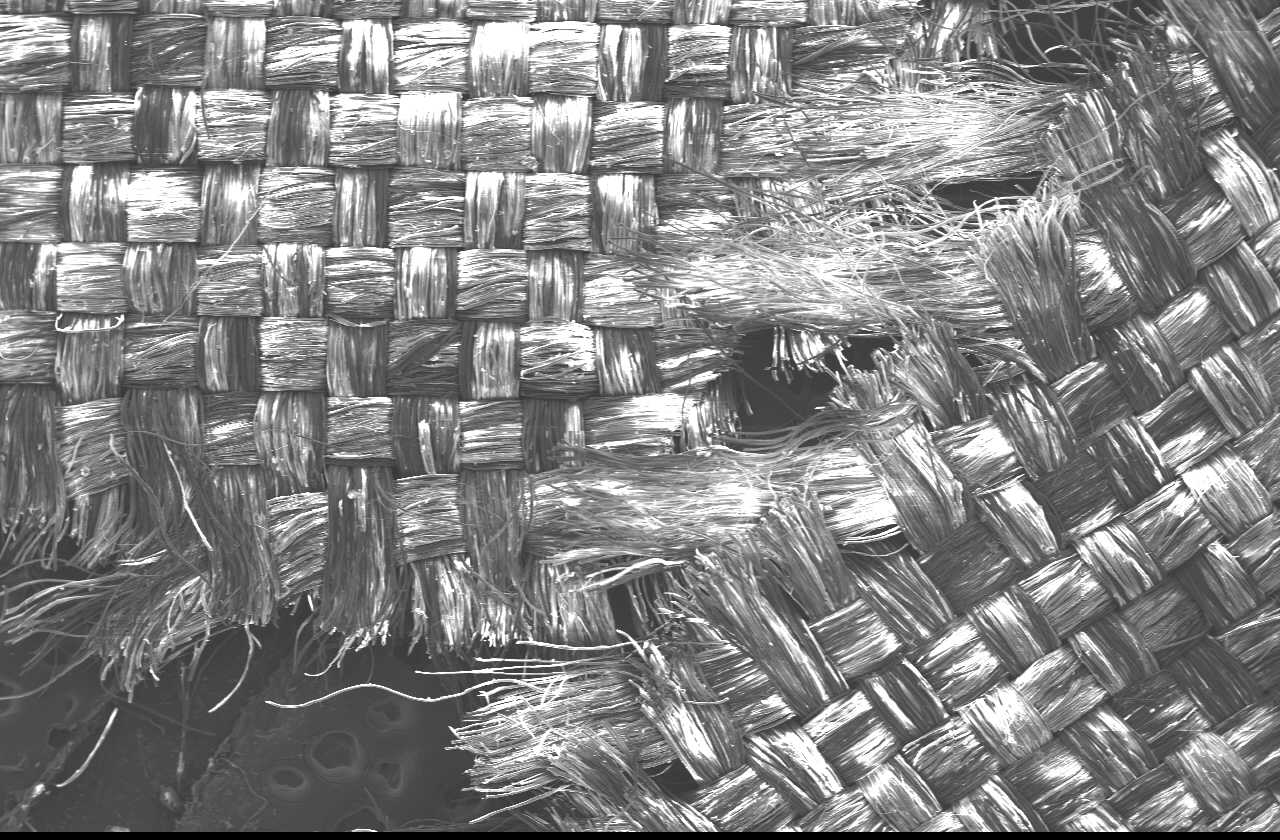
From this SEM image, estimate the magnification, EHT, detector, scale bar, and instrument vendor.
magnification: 0.02 K X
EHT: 15 kV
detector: SEI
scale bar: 1000000 nm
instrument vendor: JEOL